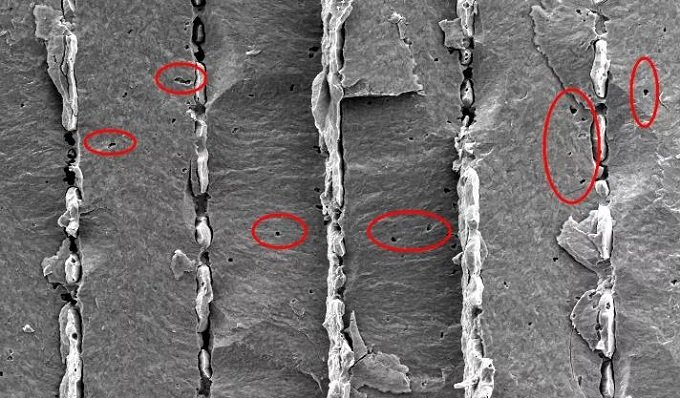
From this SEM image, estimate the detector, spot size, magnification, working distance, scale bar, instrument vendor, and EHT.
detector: SE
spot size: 3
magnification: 1 K X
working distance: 10.9 mm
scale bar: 20000 nm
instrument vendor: FEI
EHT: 8 kV